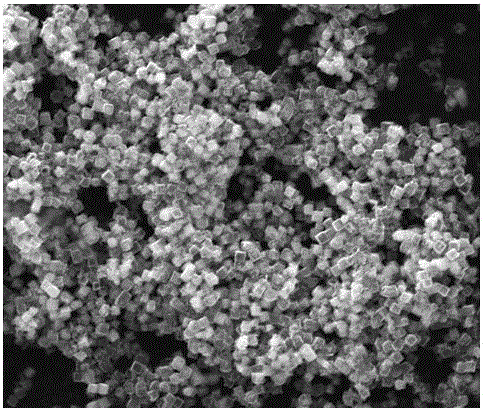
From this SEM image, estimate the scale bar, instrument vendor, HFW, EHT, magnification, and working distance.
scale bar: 10000 nm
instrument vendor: FEI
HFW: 29.8 µm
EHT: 25 kV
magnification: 10 K X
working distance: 12.4 mm